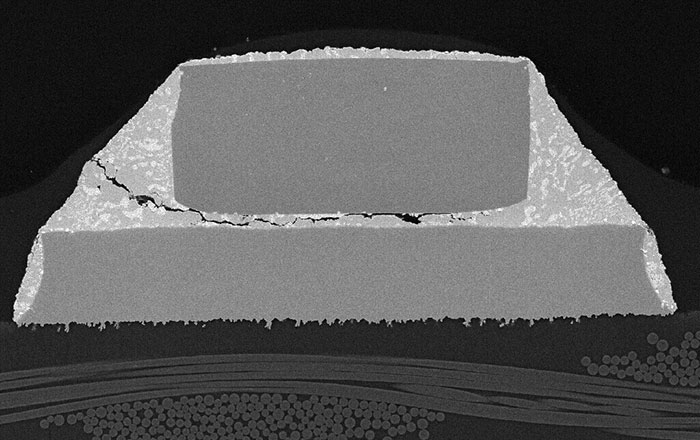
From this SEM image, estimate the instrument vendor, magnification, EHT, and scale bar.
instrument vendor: JEOL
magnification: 0.2 K X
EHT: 20 kV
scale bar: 150000 nm